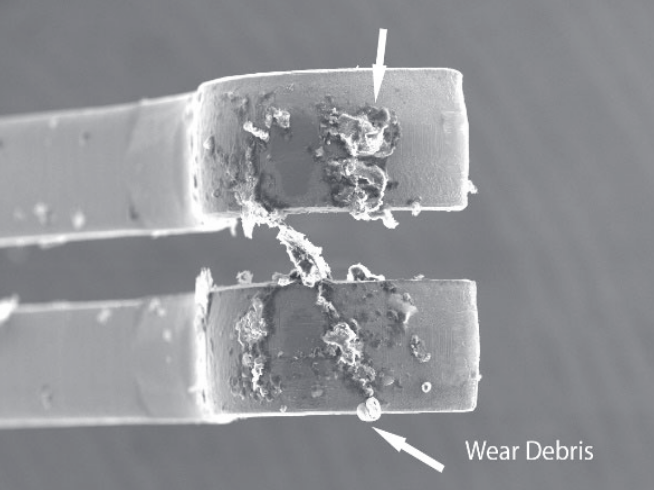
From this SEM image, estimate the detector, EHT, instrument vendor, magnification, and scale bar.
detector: SE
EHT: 20 kV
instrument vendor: Hitachi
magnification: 0.1 K X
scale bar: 300000 nm